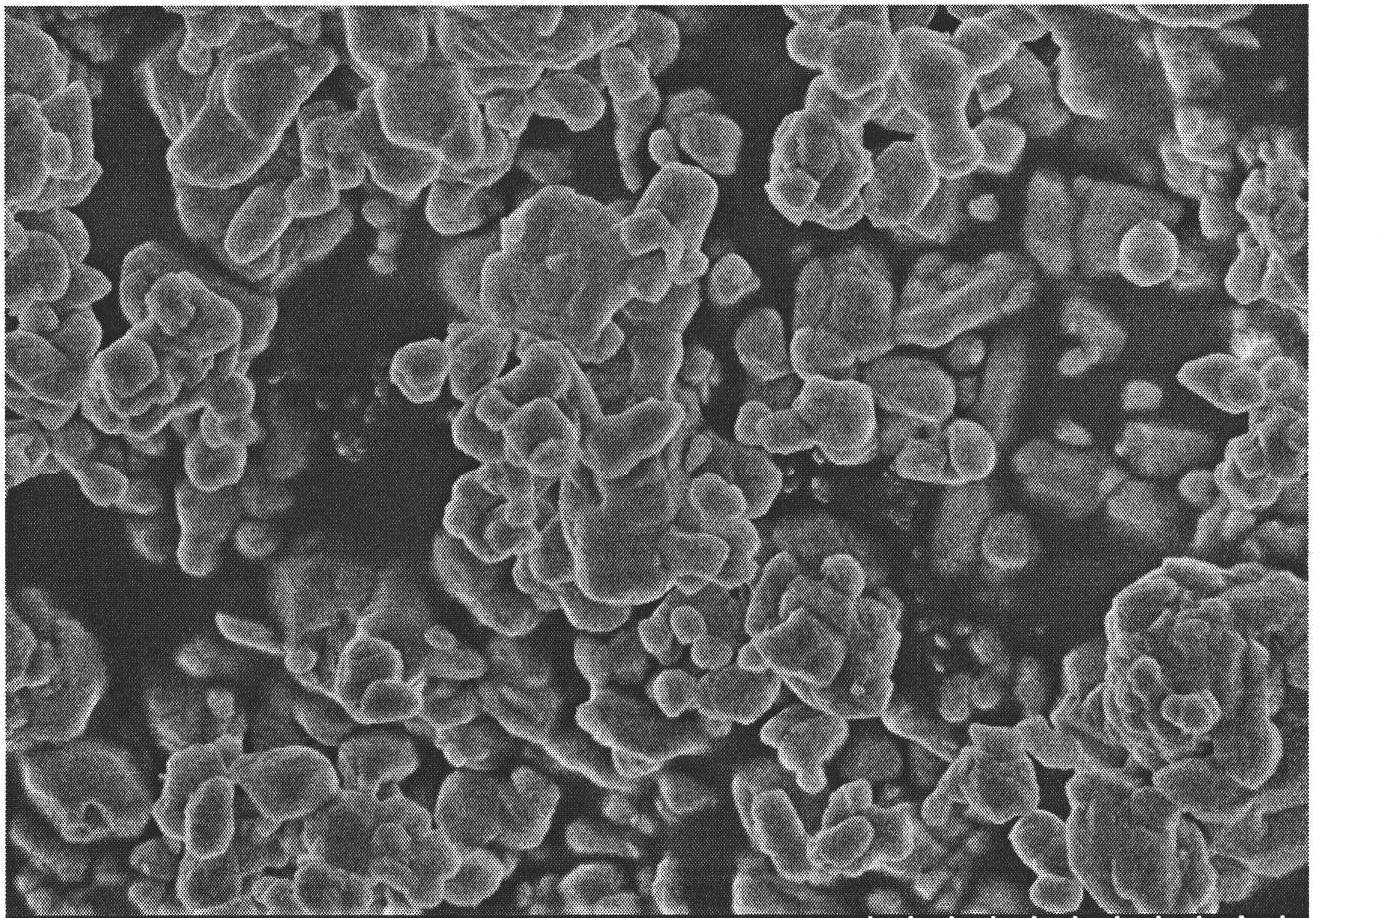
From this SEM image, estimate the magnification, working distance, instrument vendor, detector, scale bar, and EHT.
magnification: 20 K X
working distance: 8.2 mm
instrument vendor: Hitachi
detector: SE(U)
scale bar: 2000 nm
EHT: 4 kV